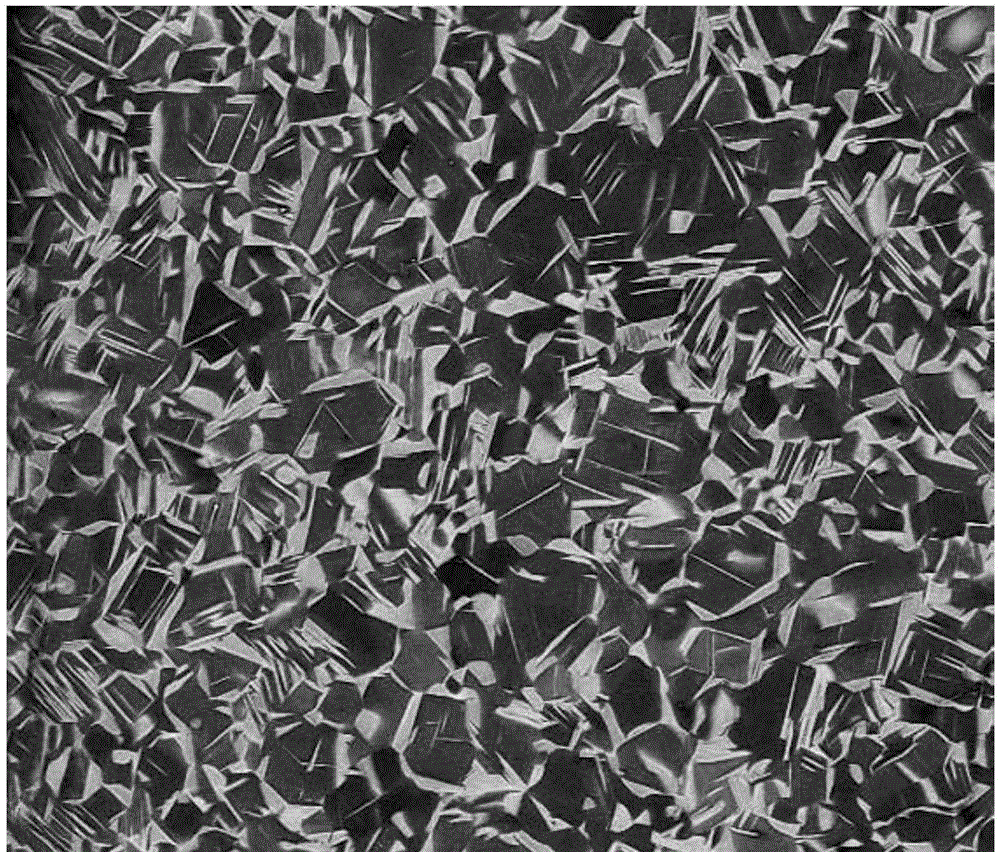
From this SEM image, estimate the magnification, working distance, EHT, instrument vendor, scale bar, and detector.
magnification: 0.5 K X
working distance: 12.4 mm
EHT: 30 kV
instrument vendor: FEI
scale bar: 200000 nm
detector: Z Cont